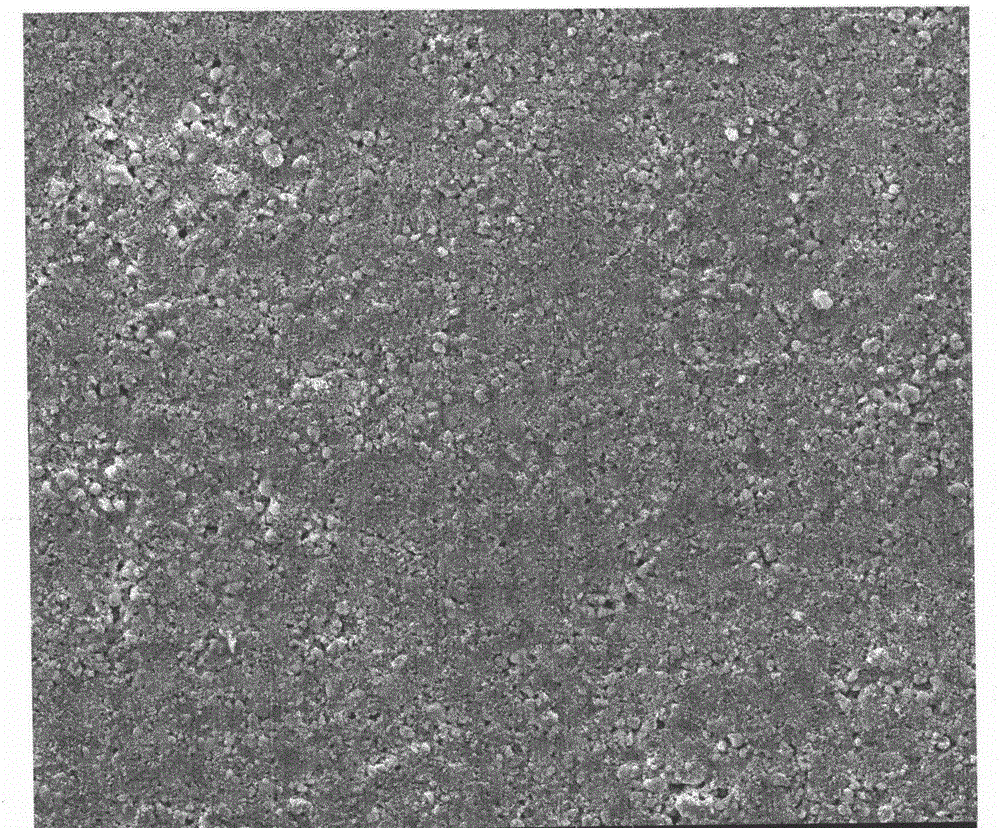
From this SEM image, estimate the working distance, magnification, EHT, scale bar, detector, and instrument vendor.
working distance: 10.1 mm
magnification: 1 K X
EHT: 20 kV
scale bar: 50000 nm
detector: ETD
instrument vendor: FEI